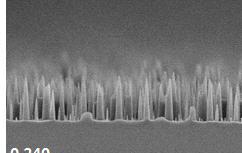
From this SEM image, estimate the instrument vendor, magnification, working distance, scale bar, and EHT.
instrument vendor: Hitachi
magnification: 10 K X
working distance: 8.2 mm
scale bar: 5000 nm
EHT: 5 kV